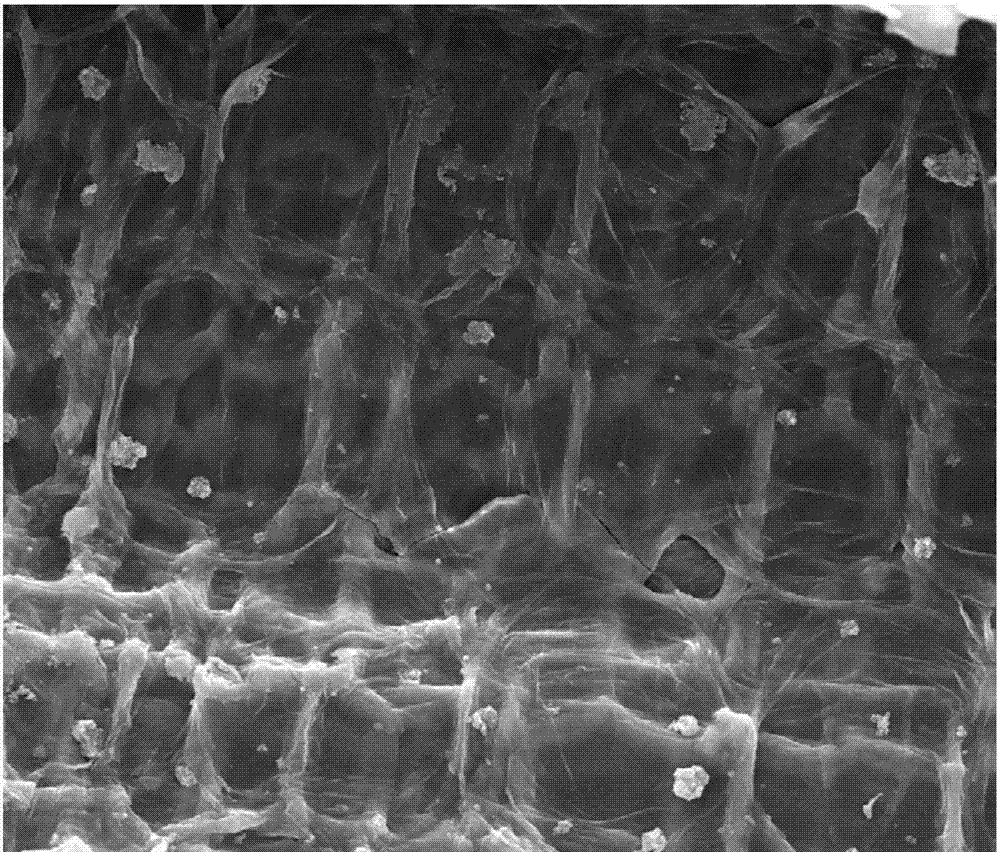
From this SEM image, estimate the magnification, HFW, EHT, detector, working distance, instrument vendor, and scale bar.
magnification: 1 K X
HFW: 298 µm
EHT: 30 kV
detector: ETD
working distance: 11 mm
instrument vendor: FEI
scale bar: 100000 nm